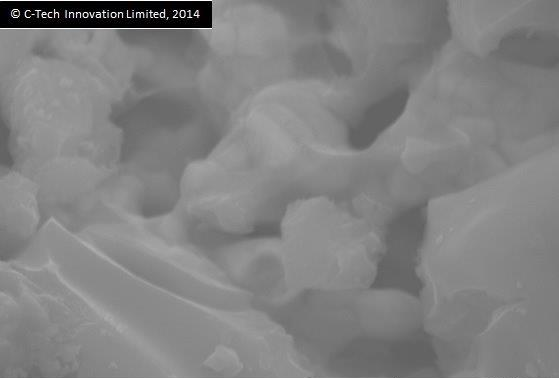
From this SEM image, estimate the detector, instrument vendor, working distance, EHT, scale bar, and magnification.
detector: SED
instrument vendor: JEOL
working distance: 6.3 mm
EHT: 15 kV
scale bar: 1000 nm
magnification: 19 K X